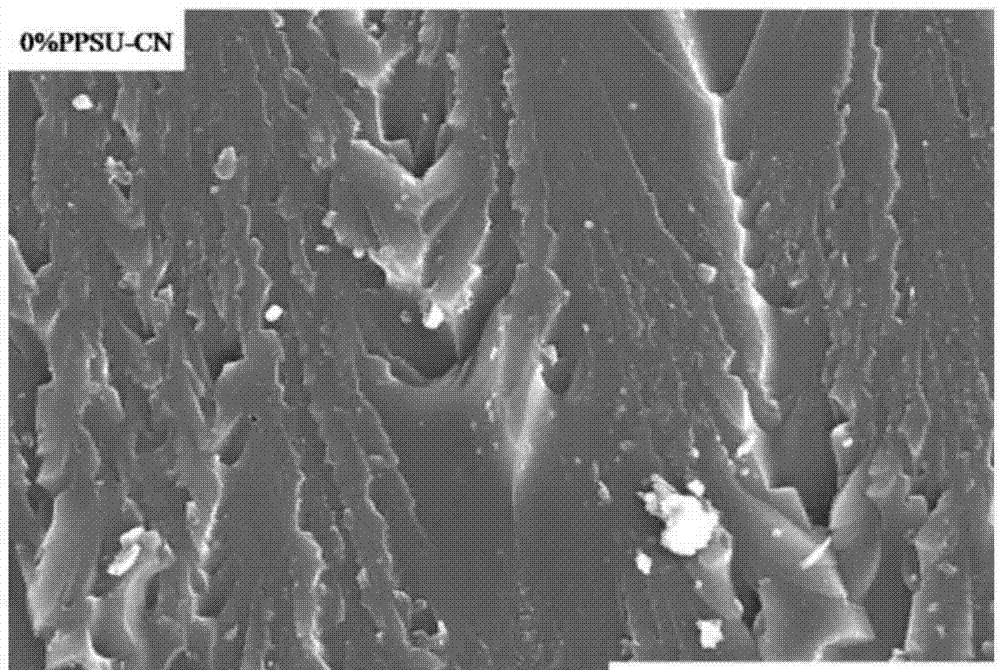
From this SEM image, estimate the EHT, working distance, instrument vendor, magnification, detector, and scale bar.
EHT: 15 kV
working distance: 6.2 mm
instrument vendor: FEI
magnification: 5 K X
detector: ETD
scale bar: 30000 nm